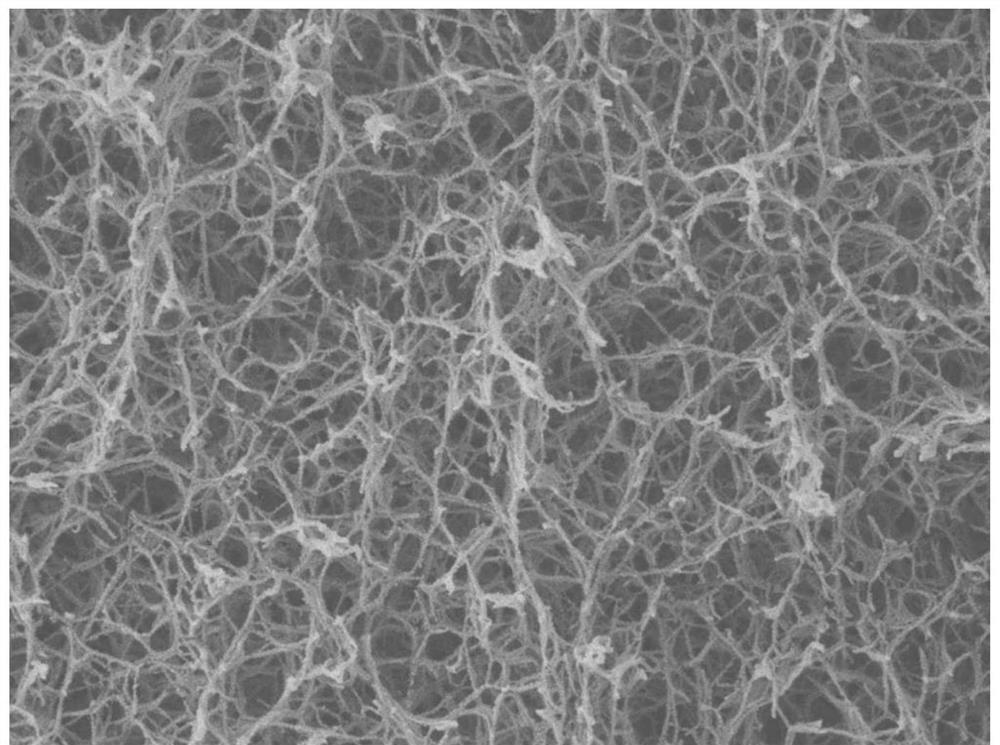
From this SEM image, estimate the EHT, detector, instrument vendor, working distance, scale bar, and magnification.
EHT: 3 kV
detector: LEI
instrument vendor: JEOL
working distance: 8.6 mm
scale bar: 1000 nm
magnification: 20 K X